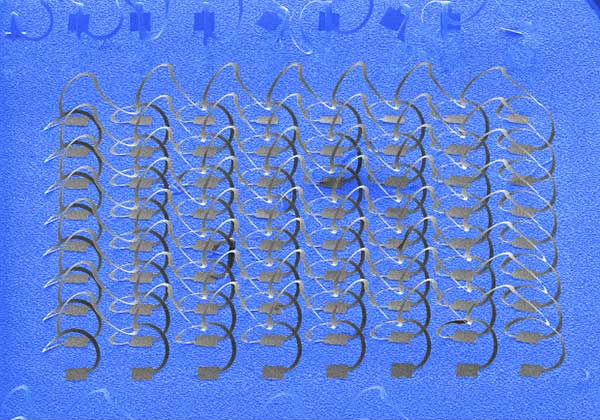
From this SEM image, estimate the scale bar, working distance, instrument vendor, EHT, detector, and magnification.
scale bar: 1000000 nm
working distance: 7.5 mm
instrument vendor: Hitachi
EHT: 10 kV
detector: SE(M)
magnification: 0.03 K X